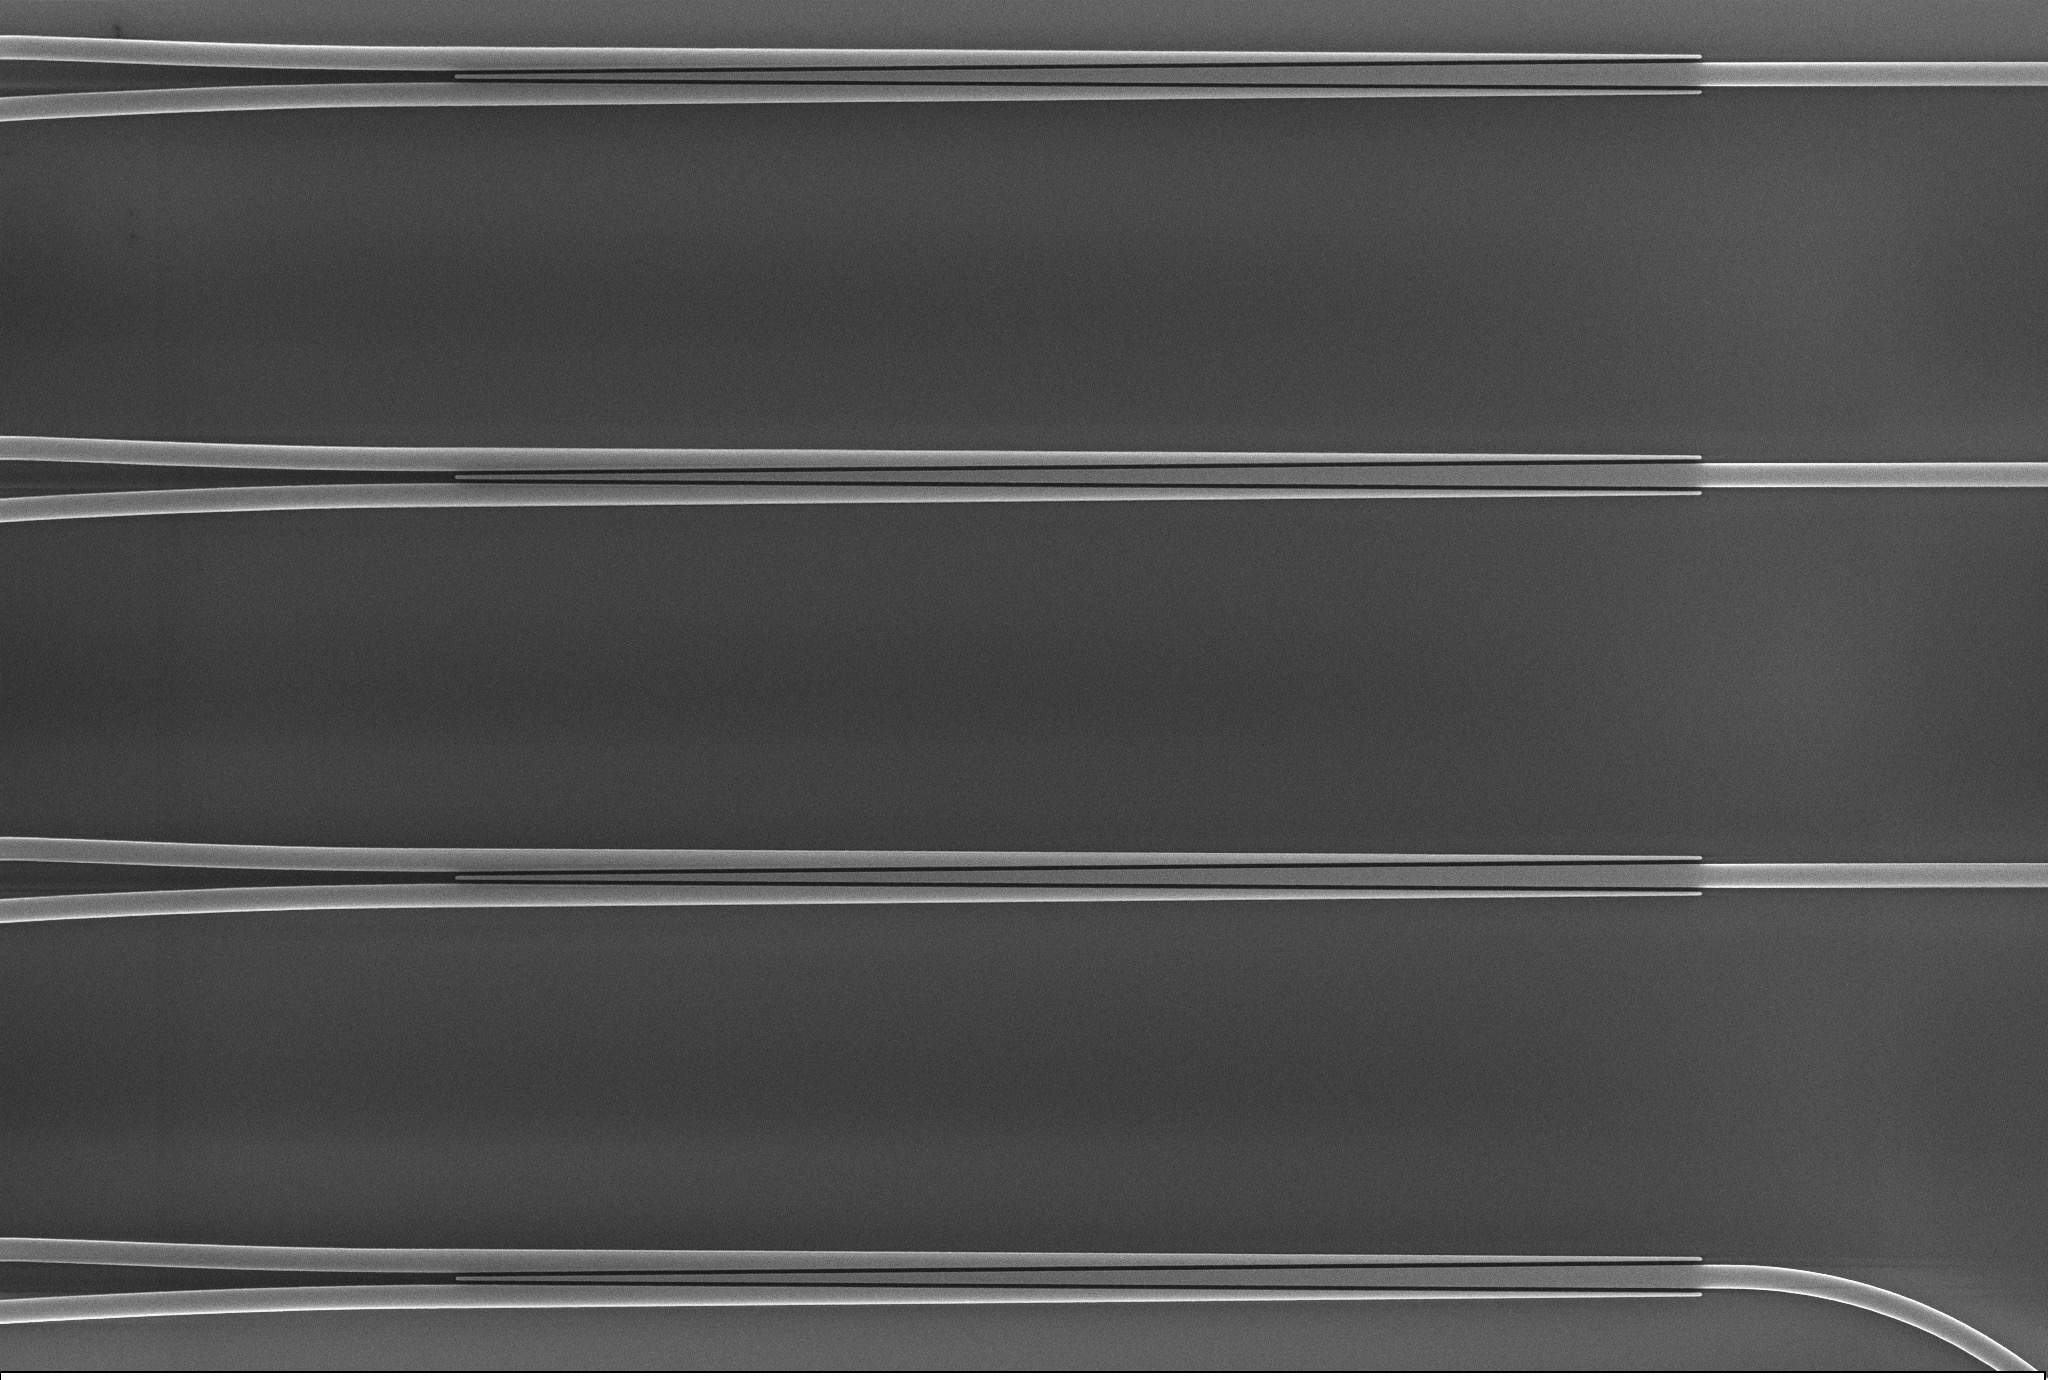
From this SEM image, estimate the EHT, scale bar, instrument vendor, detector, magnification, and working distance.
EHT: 15 kV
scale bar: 2000 nm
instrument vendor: Zeiss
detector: InLens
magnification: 2.81 K X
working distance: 6.6 mm